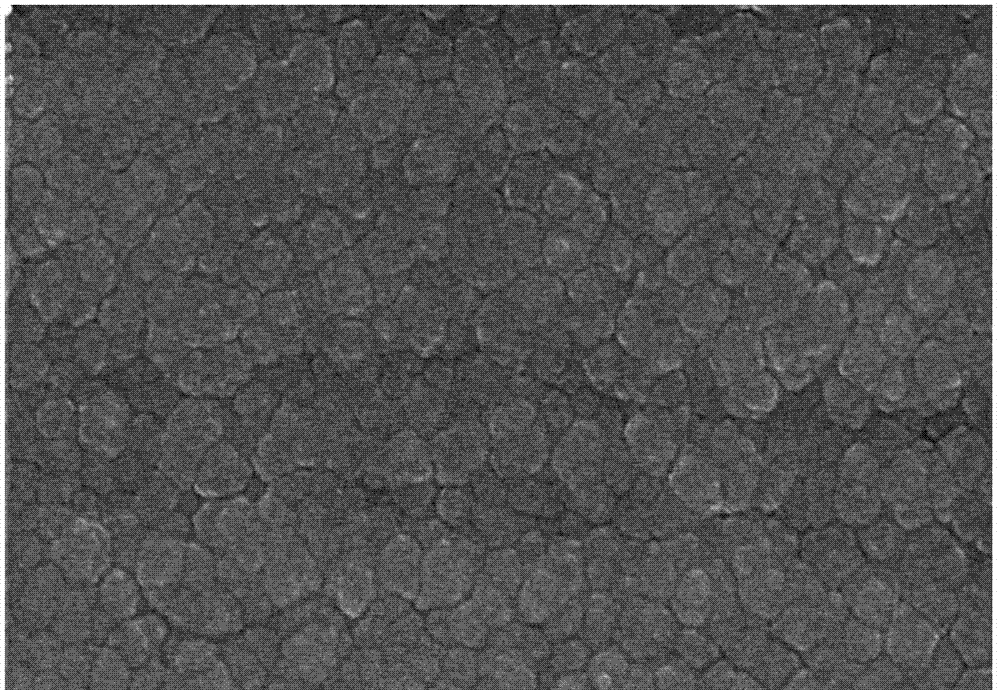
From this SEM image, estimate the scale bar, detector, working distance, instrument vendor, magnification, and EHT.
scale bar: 1000 nm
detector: SE(M)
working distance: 8 mm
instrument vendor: Hitachi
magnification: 50 K X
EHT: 10 kV